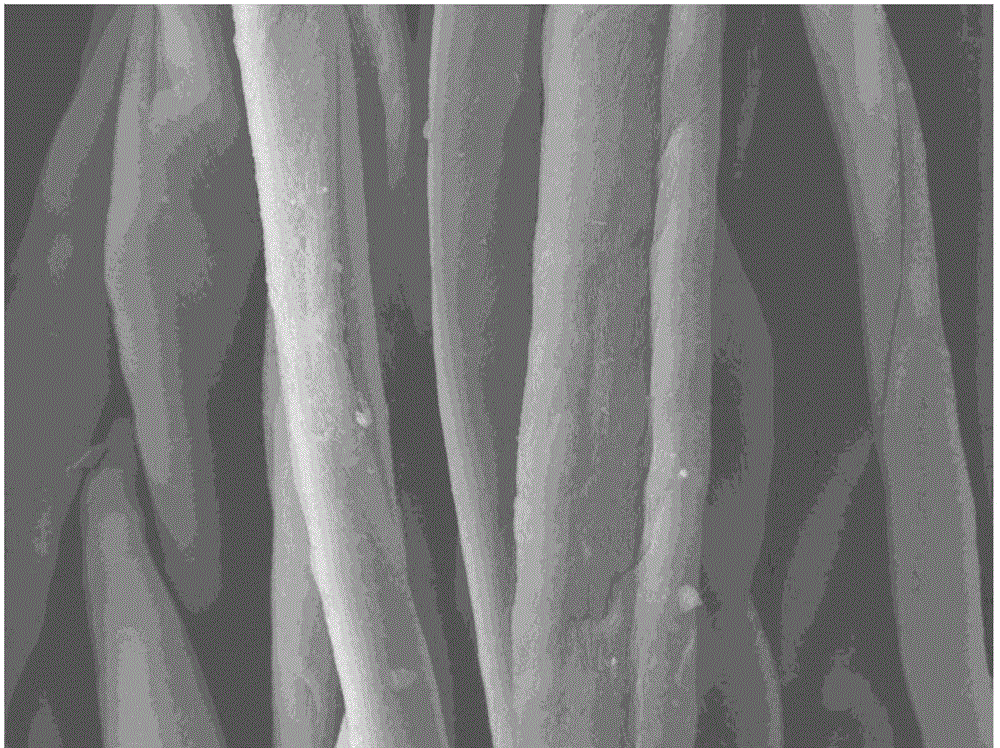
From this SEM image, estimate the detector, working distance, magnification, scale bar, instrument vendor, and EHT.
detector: LEI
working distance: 15.2 mm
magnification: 1 K X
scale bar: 10000 nm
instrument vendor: JEOL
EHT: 20 kV